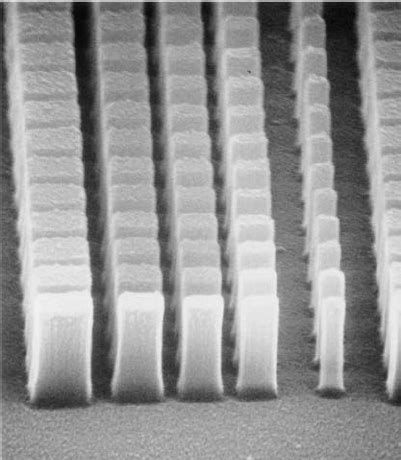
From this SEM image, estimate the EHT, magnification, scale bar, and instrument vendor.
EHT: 30 kV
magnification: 40 K X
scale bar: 750 nm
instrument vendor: JEOL